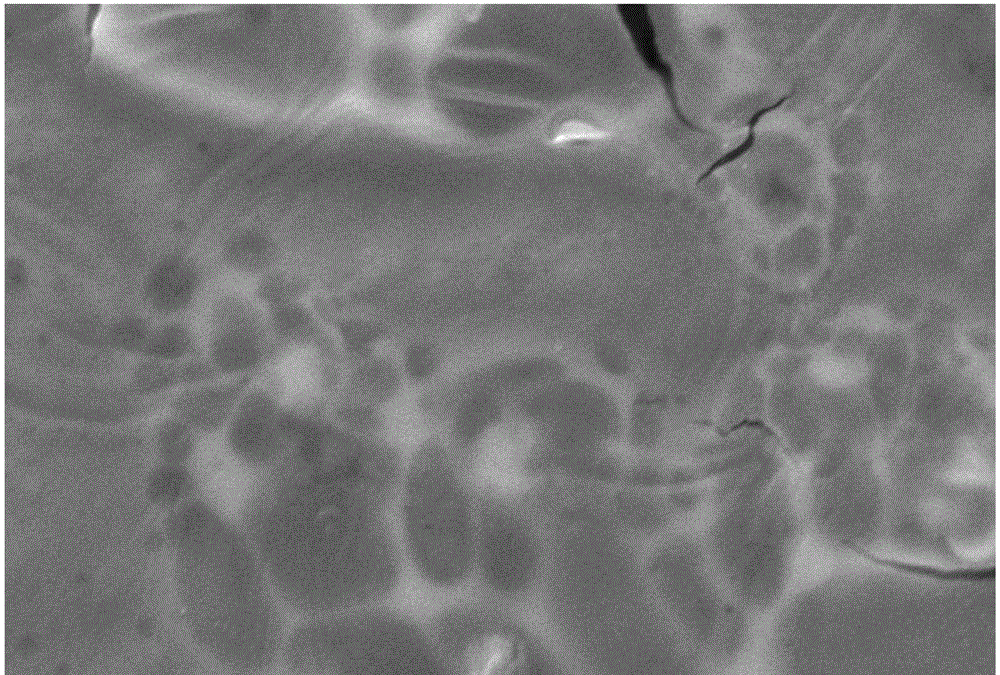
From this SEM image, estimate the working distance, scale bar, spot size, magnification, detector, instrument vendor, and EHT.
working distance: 10 mm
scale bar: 5000 nm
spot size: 50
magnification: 4.5 K X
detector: SEI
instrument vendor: JEOL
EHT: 15 kV